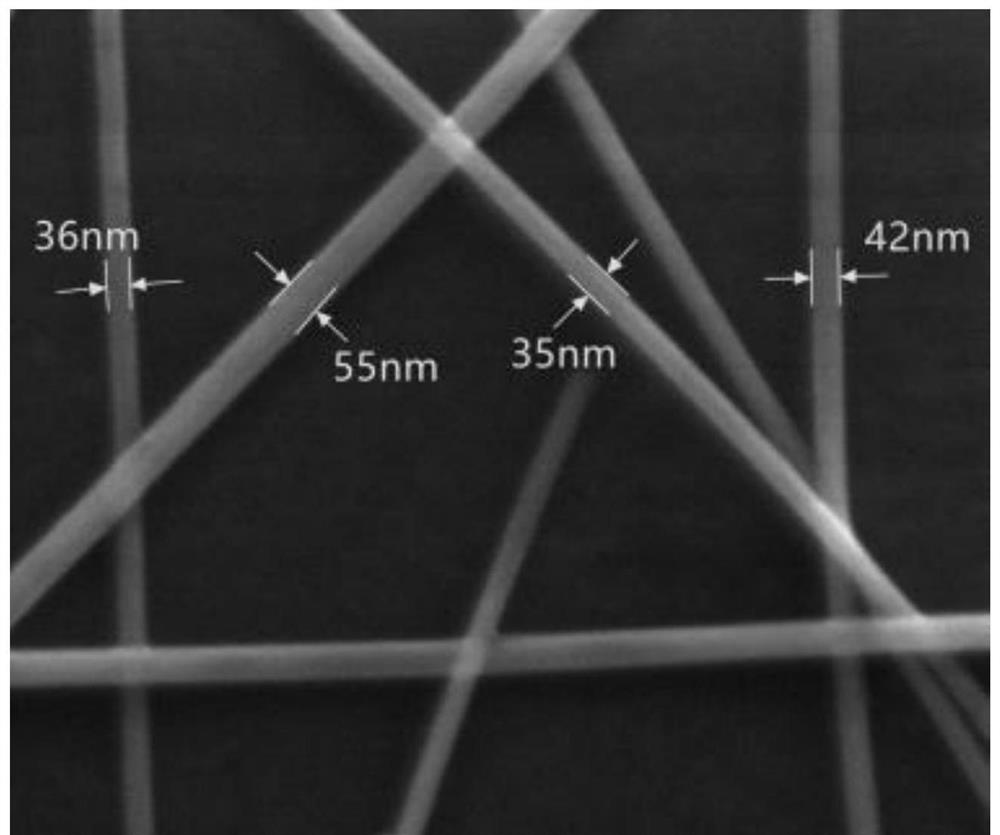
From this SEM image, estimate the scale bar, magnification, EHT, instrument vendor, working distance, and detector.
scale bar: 400 nm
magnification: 200 K X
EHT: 20 kV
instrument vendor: FEI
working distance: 6.4 mm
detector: SE